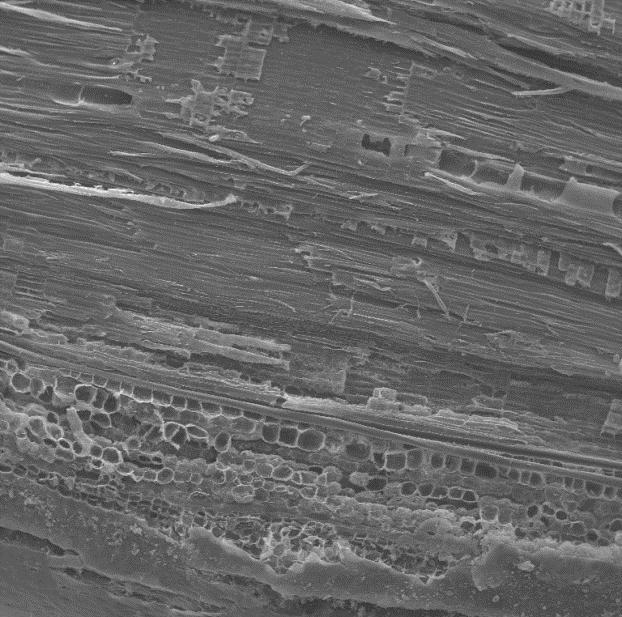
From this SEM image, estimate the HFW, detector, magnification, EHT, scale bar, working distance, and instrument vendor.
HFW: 1960 µm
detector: SE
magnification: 0.11 K X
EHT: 30 kV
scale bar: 500000 nm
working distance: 8.237 mm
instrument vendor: Tescan